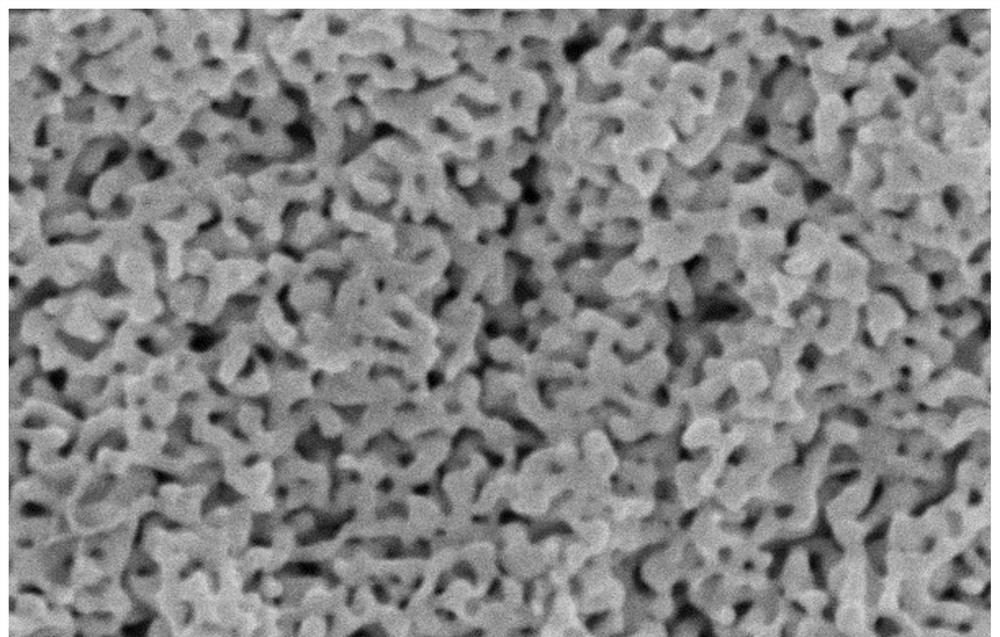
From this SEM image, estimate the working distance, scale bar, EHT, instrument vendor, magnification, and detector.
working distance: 9.6 mm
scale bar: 1000 nm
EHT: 10 kV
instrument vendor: Hitachi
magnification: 50 K X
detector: SE(U)